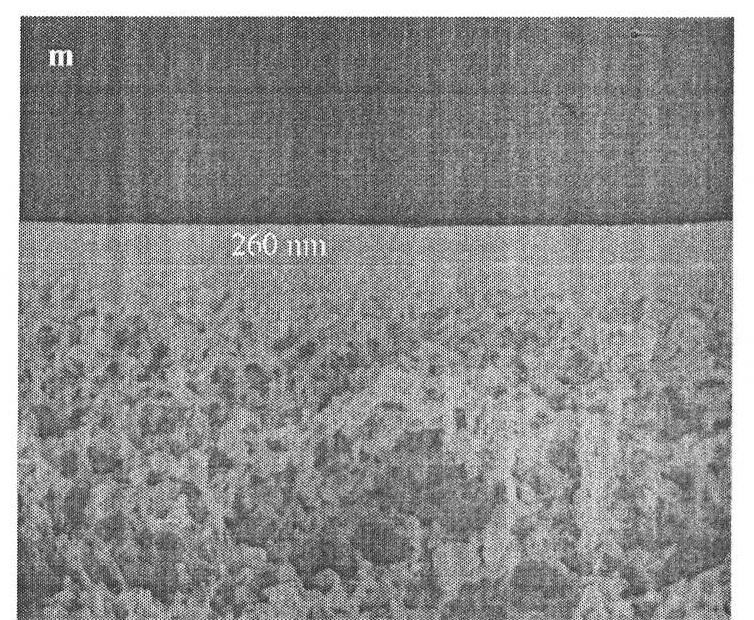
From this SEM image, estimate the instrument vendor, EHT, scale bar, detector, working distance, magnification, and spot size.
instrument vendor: FEI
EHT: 10 kV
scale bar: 1000 nm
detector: ETD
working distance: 5.2 mm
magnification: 50 K X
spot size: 3